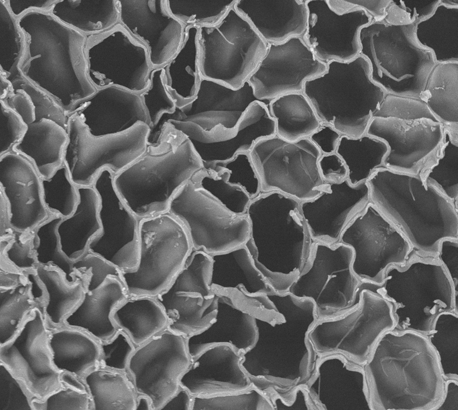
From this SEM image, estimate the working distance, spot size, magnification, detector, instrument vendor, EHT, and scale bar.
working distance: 9.9 mm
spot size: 3.5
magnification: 0.6 K X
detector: BSE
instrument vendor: FEI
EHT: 15 kV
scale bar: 50000 nm